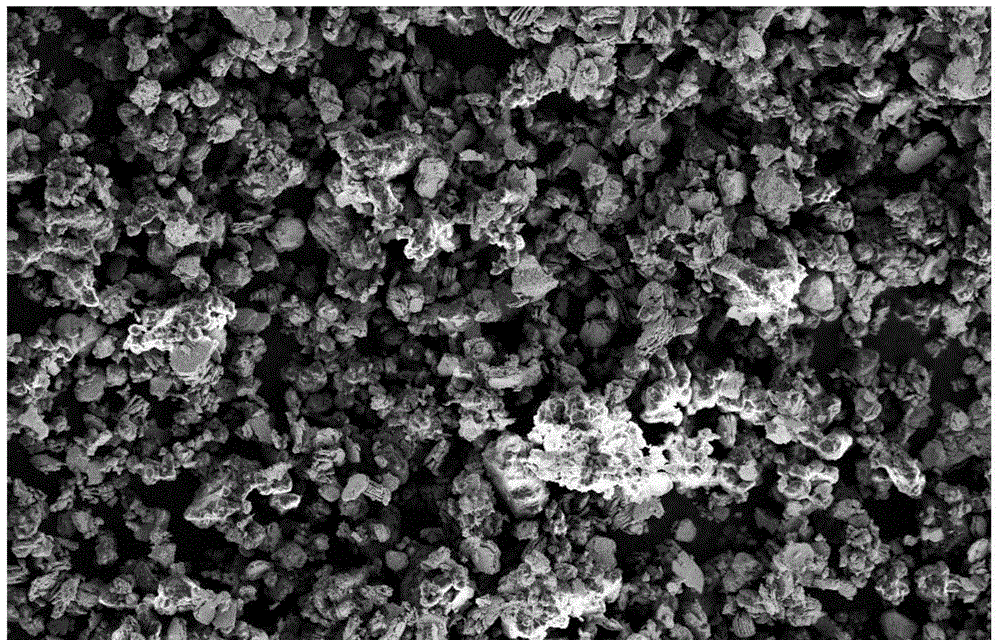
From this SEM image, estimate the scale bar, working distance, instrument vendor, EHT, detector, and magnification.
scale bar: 10000 nm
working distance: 7.5 mm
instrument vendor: Zeiss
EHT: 5 kV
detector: SE2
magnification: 1 K X